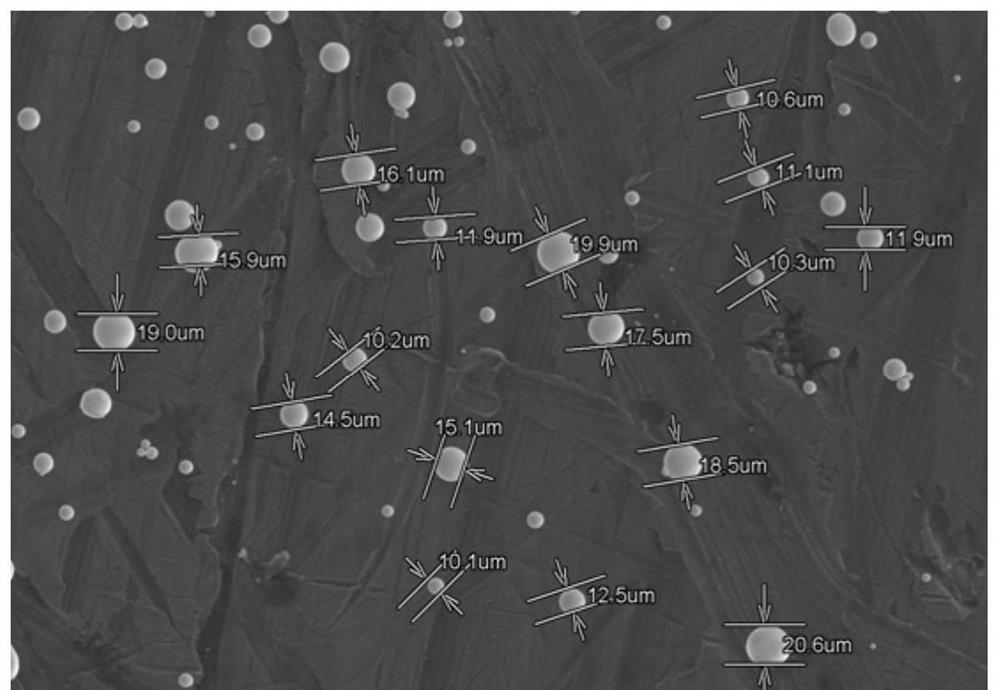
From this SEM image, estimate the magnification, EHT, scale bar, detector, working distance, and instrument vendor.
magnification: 0.25 K X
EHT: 15 kV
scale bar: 200000 nm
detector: SE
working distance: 24.1 mm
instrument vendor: Hitachi